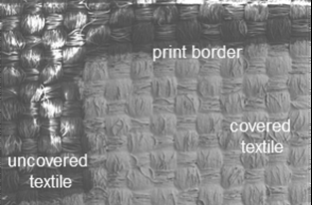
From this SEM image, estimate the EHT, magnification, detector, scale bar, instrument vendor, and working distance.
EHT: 15 kV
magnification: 0.03 K X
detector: SE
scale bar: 1000000 nm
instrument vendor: Hitachi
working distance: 32.1 mm